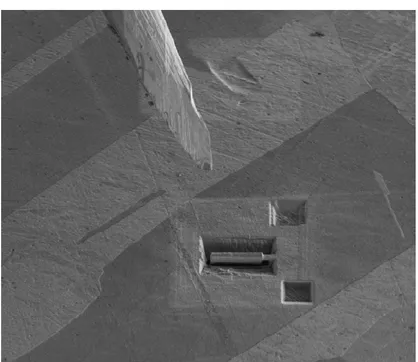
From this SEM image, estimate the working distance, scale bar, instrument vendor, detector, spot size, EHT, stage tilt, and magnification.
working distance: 5.308 mm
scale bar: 50000 nm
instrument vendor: FEI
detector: SED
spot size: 5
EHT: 2 kV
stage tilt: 52°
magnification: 2 K X